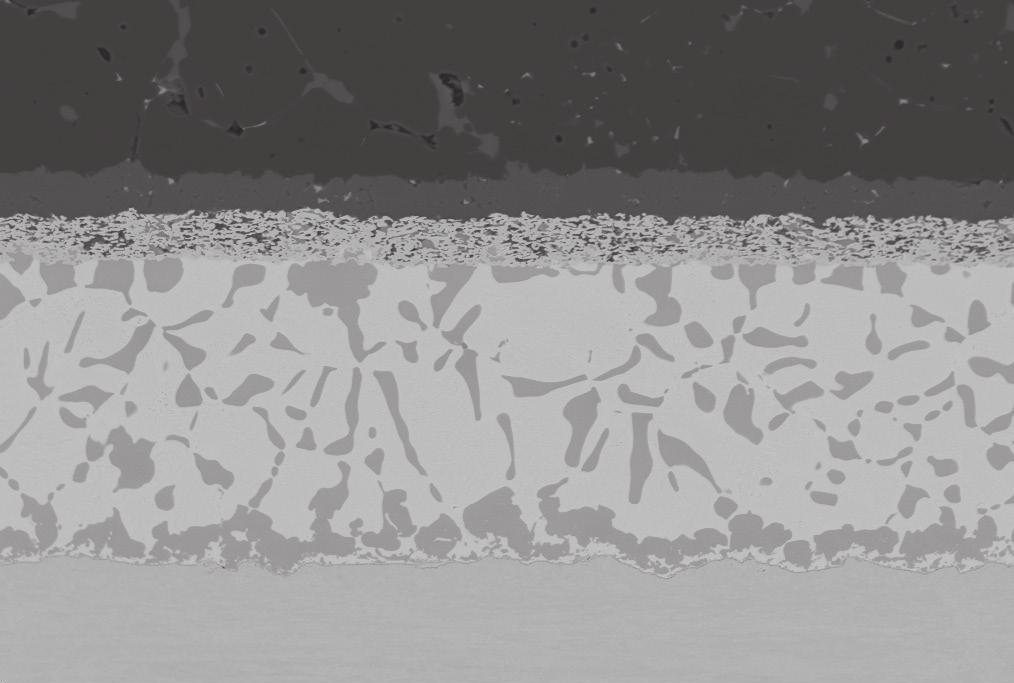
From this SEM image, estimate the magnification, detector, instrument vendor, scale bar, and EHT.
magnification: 1.3 K X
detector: QBSD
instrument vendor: Zeiss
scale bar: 30000 nm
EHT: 20 kV